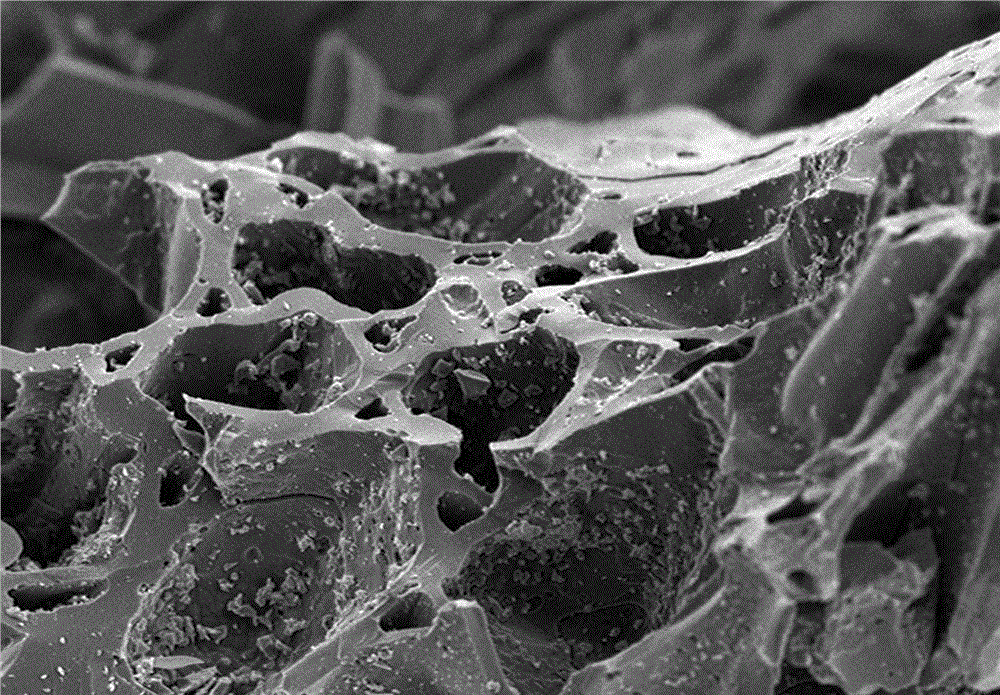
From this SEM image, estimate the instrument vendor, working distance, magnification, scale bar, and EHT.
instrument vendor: Hitachi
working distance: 14.7 mm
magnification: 1 K X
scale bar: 10000 nm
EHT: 5 kV